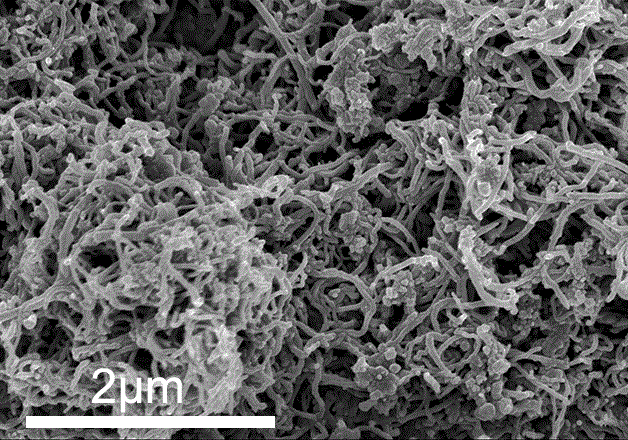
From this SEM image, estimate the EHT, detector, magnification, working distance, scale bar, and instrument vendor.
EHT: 3 kV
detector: SE(M)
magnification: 25 K X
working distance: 8.6 mm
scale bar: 2000 nm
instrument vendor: Hitachi